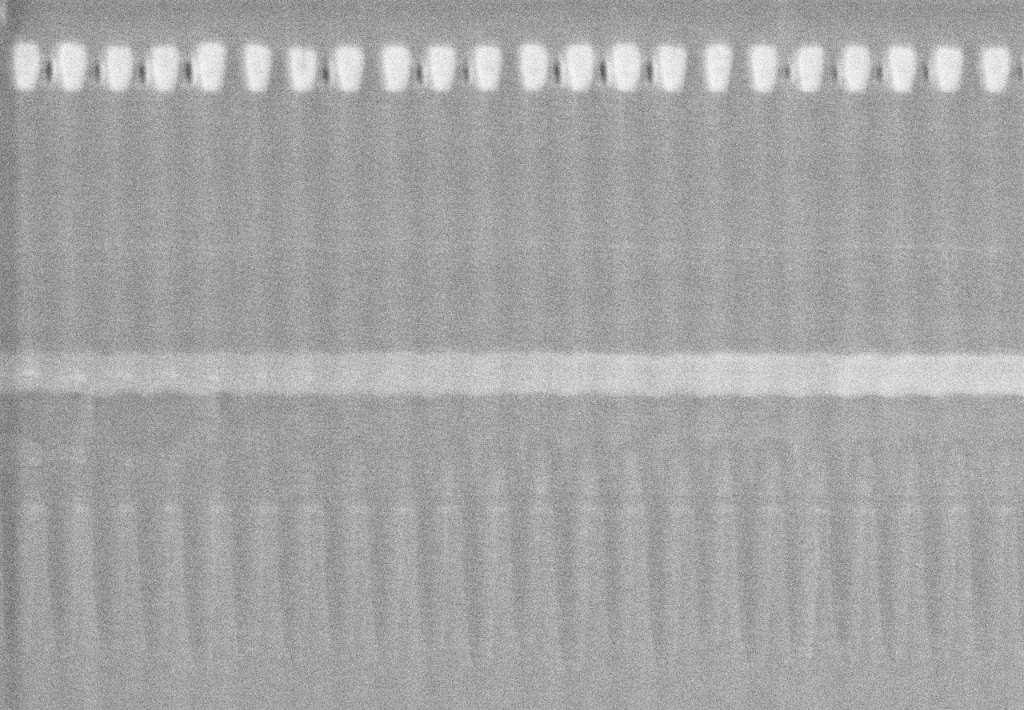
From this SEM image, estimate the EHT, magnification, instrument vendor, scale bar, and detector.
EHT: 200 kV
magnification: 100 K X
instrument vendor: Hitachi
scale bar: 300 nm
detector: SE